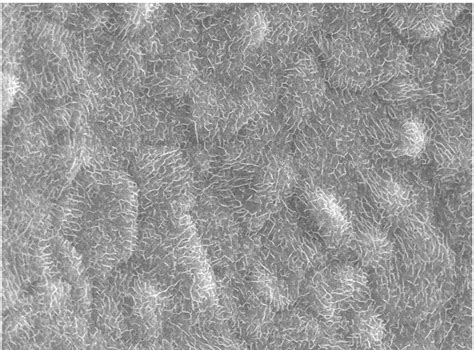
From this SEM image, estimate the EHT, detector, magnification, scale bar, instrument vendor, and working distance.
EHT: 20 kV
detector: SEI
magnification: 5 K X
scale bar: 1000 nm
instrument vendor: JEOL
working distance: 10 mm